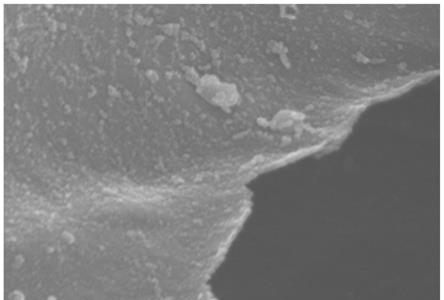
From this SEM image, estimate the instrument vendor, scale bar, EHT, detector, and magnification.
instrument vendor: Hitachi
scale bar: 500 nm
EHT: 8 kV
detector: SE(M)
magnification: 100 K X